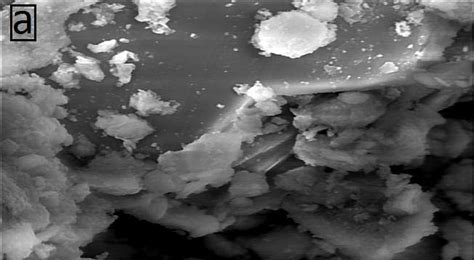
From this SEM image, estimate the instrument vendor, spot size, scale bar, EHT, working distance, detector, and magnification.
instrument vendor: FEI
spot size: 4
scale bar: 3000 nm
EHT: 7 kV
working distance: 9.3 mm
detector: LFD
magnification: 25 K X